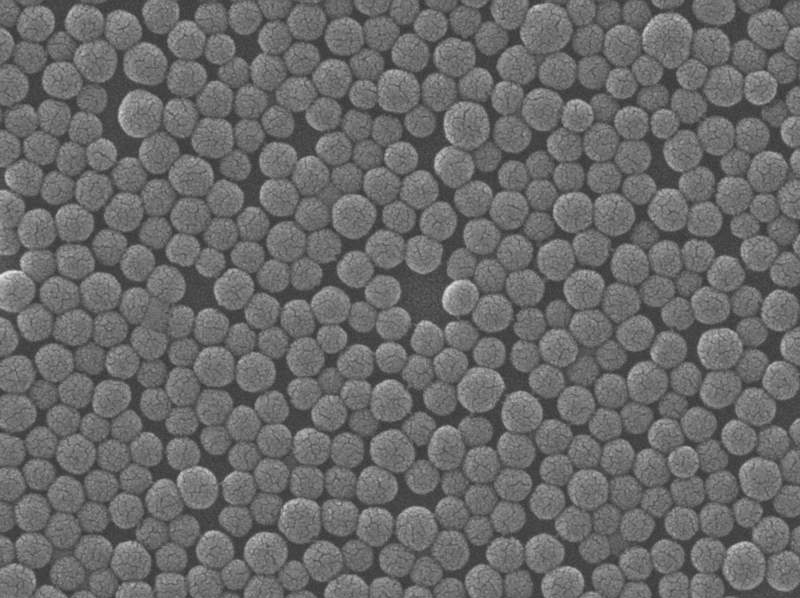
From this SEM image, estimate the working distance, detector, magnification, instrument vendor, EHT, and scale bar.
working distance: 4.9 mm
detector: SEI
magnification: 55 K X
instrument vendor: JEOL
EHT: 20 kV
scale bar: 100 nm